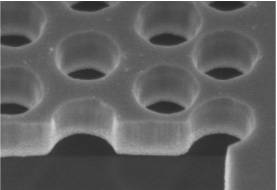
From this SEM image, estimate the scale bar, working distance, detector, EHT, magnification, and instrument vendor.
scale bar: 500 nm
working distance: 4 mm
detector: SE(M)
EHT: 2 kV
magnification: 100 K X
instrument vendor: Hitachi